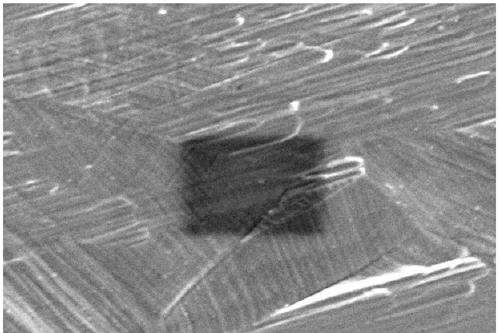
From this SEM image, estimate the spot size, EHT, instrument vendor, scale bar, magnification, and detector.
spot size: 50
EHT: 10 kV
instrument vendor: JEOL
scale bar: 5000 nm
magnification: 5 K X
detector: SEI